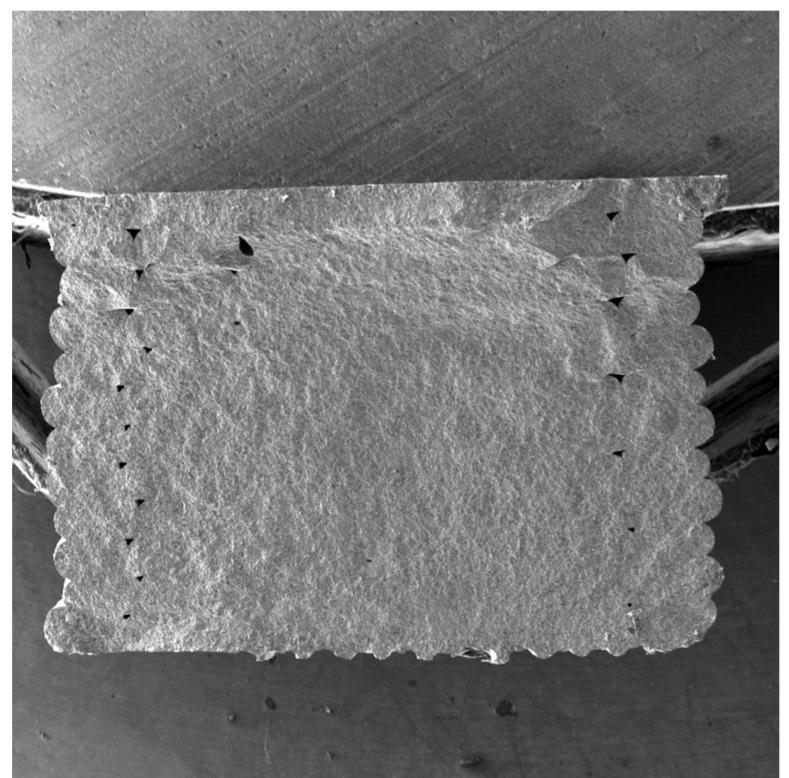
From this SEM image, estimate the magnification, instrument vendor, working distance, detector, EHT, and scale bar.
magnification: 0.067 K X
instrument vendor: Tescan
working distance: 14.98 mm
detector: SE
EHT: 10 kV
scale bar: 500000 nm